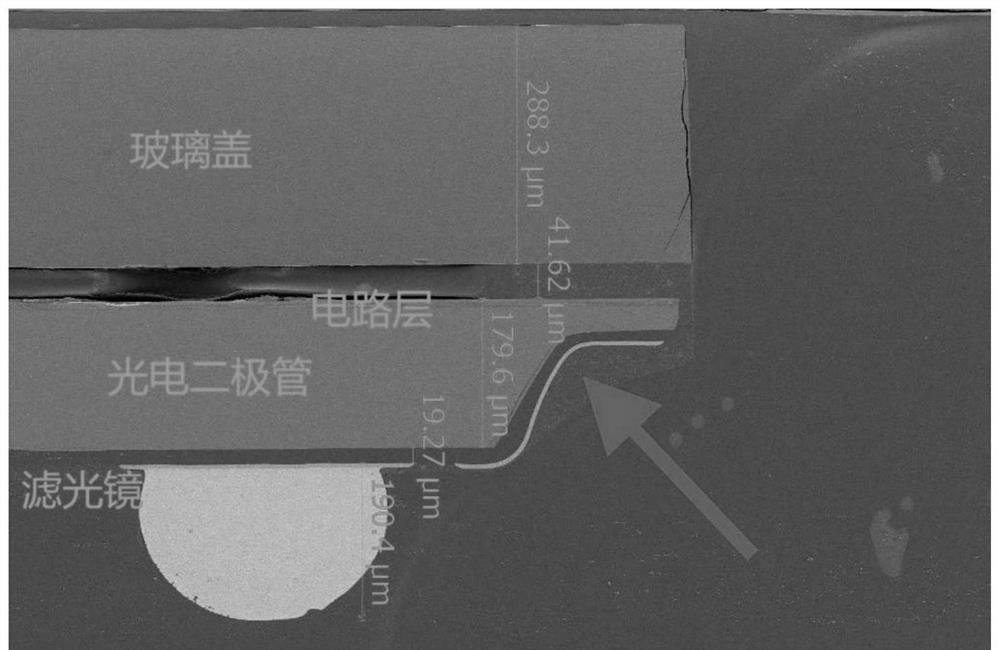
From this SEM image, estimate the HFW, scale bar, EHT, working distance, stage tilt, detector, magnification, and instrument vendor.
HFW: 1180 µm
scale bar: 300000 nm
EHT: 5 kV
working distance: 10 mm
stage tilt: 0°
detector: ETD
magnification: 0.35 K X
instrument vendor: Thermo